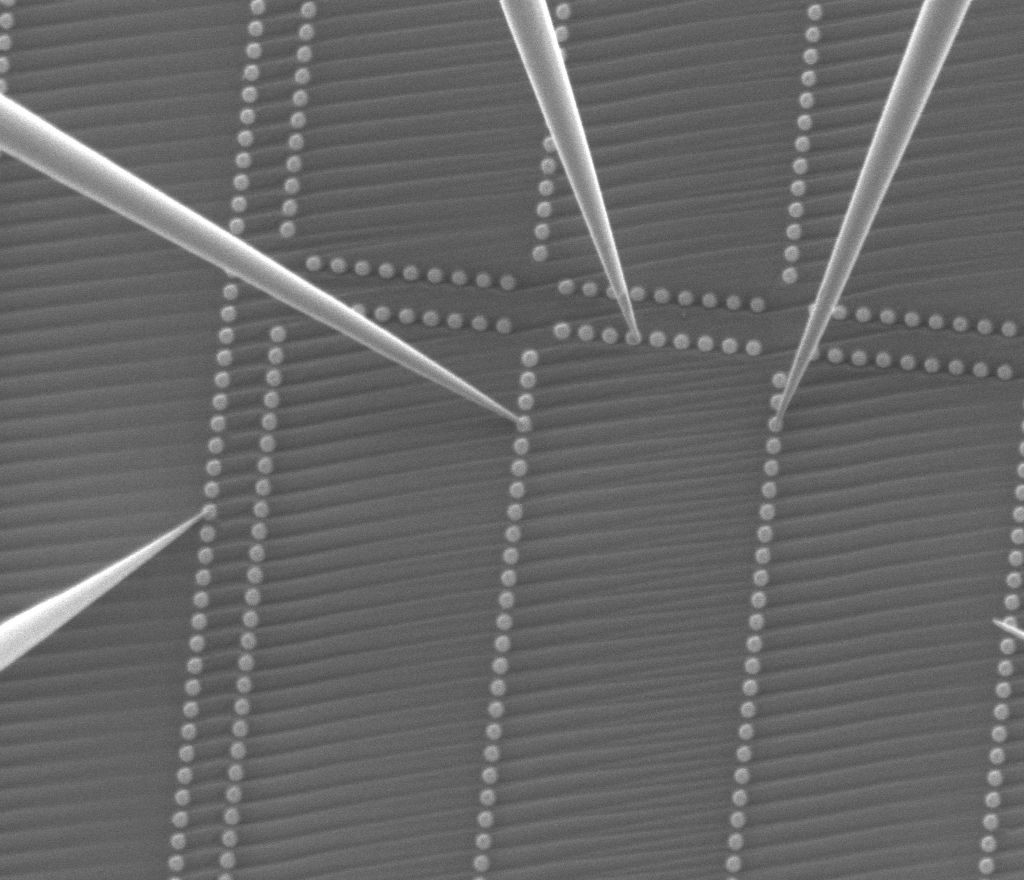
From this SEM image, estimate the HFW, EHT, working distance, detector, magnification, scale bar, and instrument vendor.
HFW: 17.8 µm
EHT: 1 kV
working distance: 8 mm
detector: ETD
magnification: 16.727 K X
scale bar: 5000 nm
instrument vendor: FEI